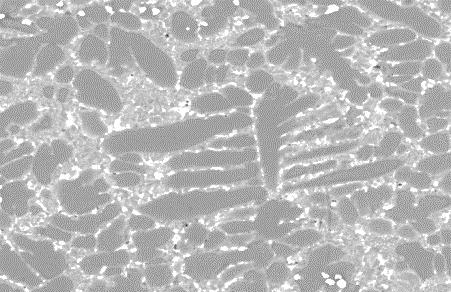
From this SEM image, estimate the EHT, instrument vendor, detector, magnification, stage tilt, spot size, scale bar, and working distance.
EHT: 25 kV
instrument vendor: FEI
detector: BSED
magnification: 4 K X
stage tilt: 0°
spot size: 5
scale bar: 30000 nm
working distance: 8.4 mm